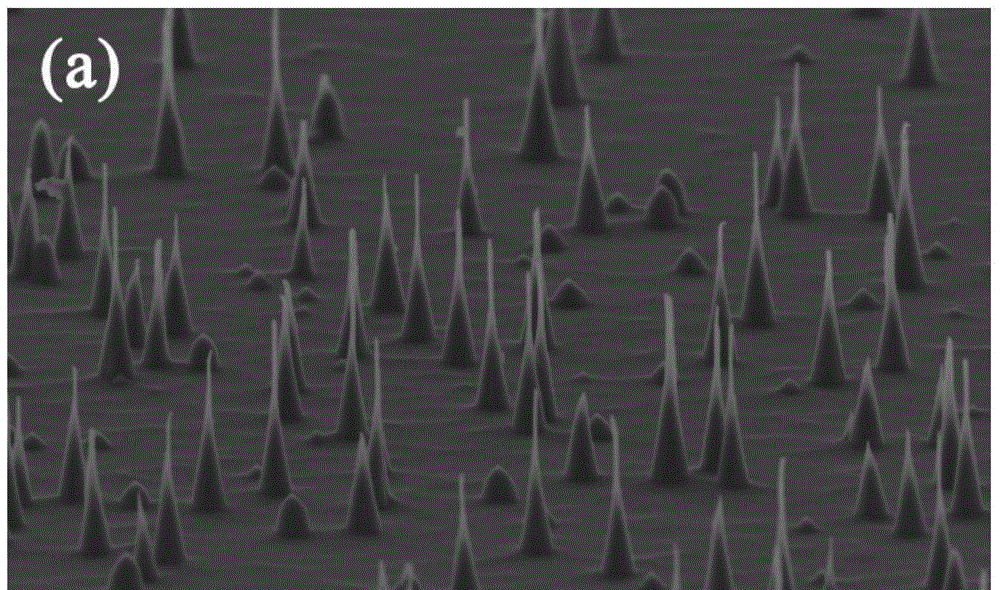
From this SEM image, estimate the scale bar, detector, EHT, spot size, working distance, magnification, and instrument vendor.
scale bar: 5000 nm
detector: SE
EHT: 5 kV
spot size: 3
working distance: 10.2 mm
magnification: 15 K X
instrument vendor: FEI